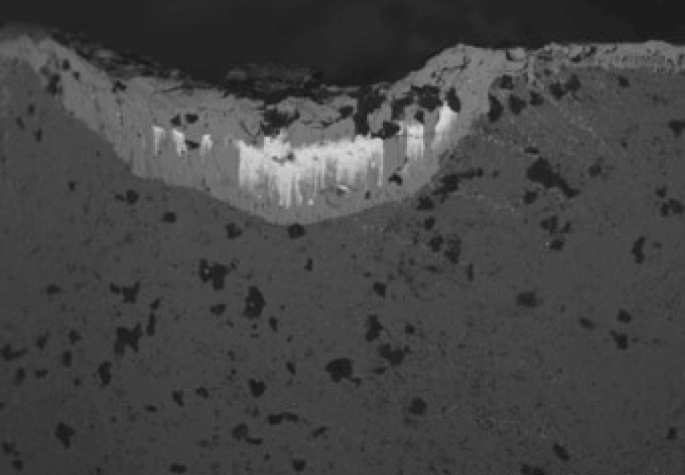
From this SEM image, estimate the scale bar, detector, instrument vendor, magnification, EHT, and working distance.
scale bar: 500000 nm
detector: BSE3D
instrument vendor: Hitachi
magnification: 0.1 K X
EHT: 15 kV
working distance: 11.1 mm